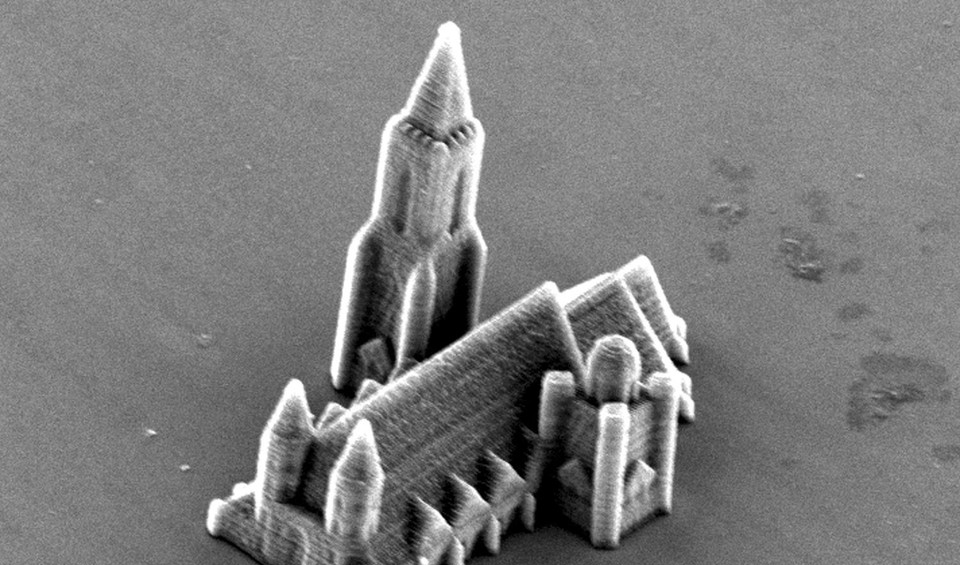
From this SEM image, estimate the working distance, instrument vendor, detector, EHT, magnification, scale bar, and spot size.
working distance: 27.5 mm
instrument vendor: FEI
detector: SE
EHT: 15 kV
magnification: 0.887 K X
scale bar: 20000 nm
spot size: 4